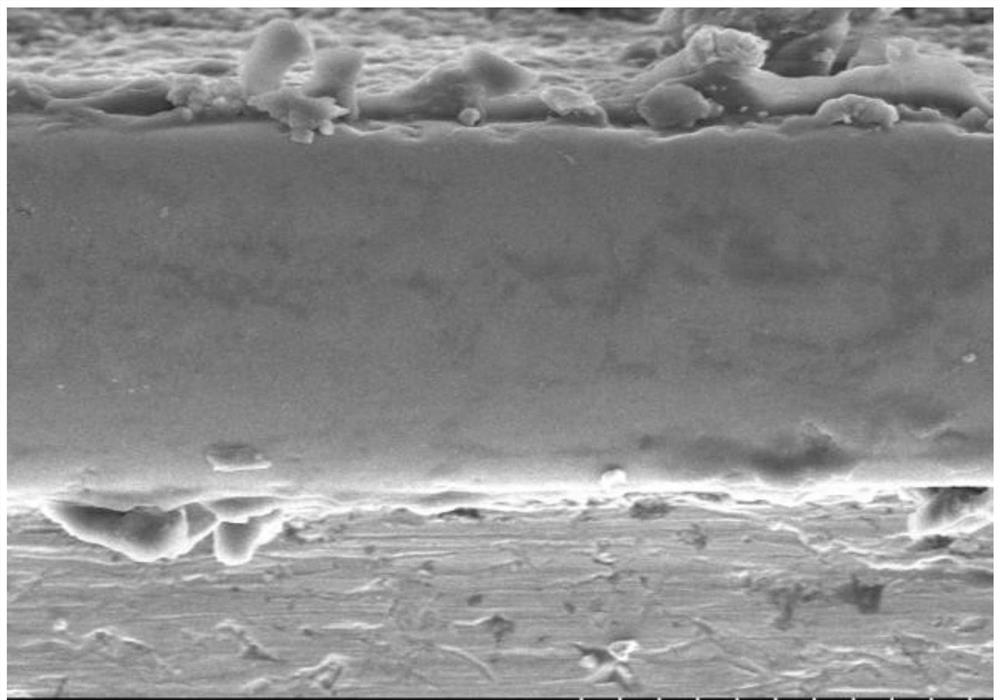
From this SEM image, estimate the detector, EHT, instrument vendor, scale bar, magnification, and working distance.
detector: SE(UL)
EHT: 5 kV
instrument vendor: Hitachi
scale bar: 10000 nm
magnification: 5 K X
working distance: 10.8 mm